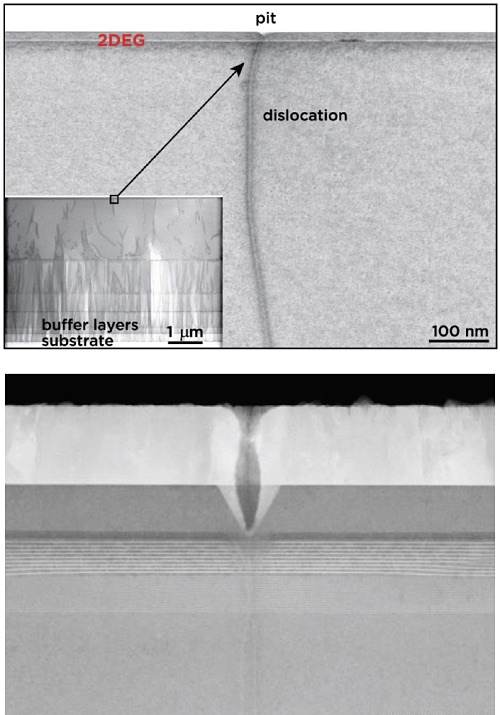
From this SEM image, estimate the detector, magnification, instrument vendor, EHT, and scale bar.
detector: ZC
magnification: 130 K X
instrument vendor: Hitachi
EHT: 200 kV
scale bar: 200 nm